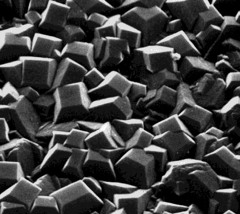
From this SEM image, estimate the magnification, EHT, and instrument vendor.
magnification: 8 K X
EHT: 25 kV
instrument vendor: Hitachi